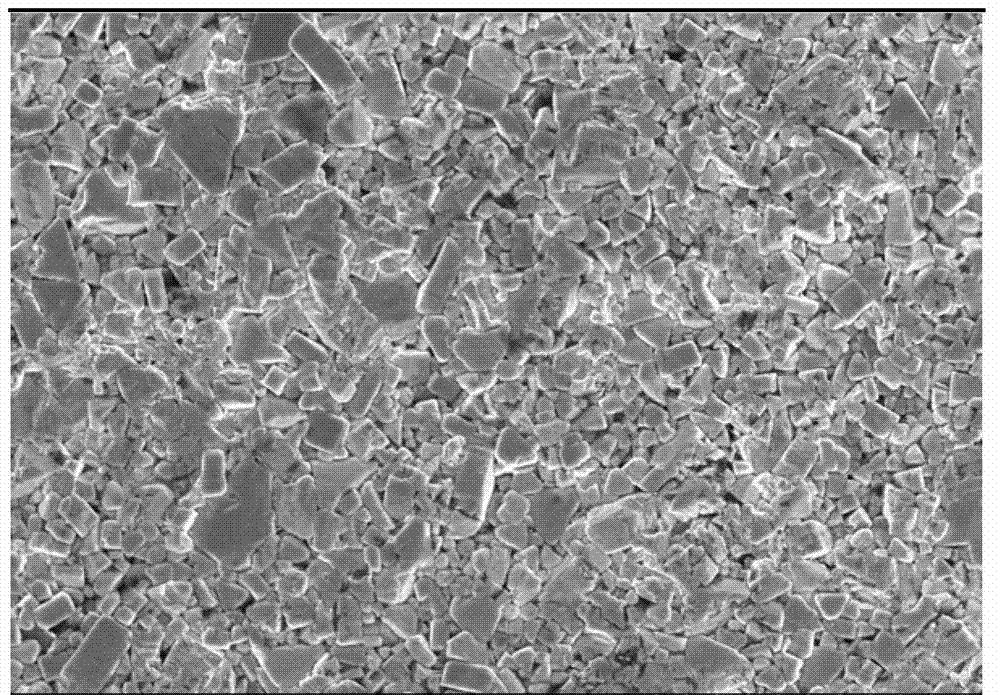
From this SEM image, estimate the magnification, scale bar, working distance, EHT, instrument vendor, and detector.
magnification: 2 K X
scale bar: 20000 nm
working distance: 8.1 mm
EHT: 15 kV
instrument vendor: Hitachi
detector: SE(M)